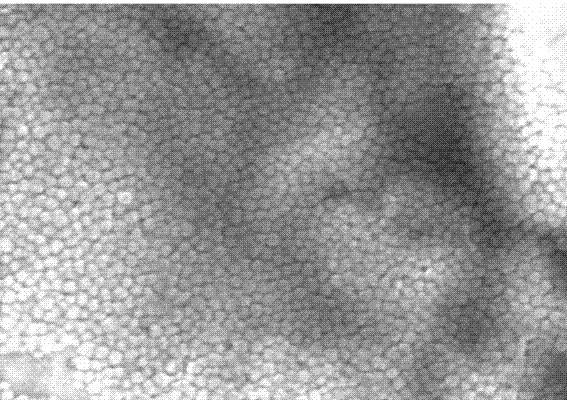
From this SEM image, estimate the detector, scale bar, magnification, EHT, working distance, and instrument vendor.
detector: SE(M)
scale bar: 500 nm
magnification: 100 K X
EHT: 10 kV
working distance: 7.7 mm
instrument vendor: Hitachi